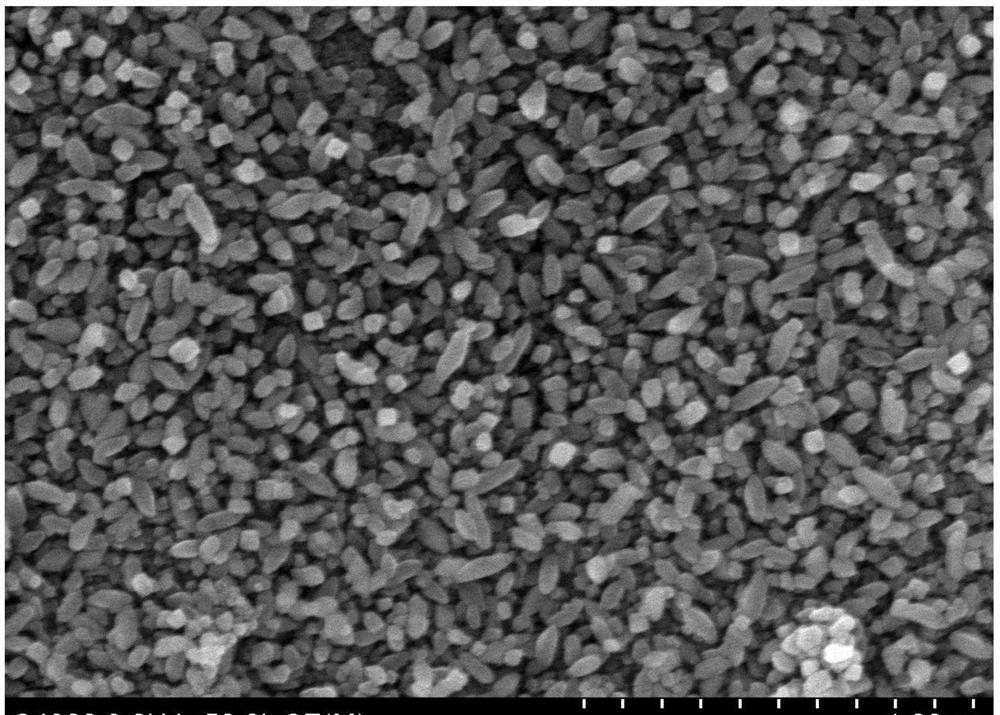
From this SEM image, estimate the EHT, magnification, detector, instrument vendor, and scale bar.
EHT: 8 kV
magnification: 50 K X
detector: SE(M)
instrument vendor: Hitachi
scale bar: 1000 nm